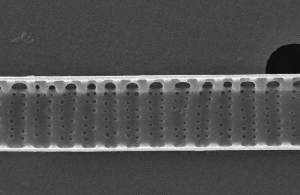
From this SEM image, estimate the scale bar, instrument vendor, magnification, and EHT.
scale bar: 1000 nm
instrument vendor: JEOL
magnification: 10 K X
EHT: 10 kV